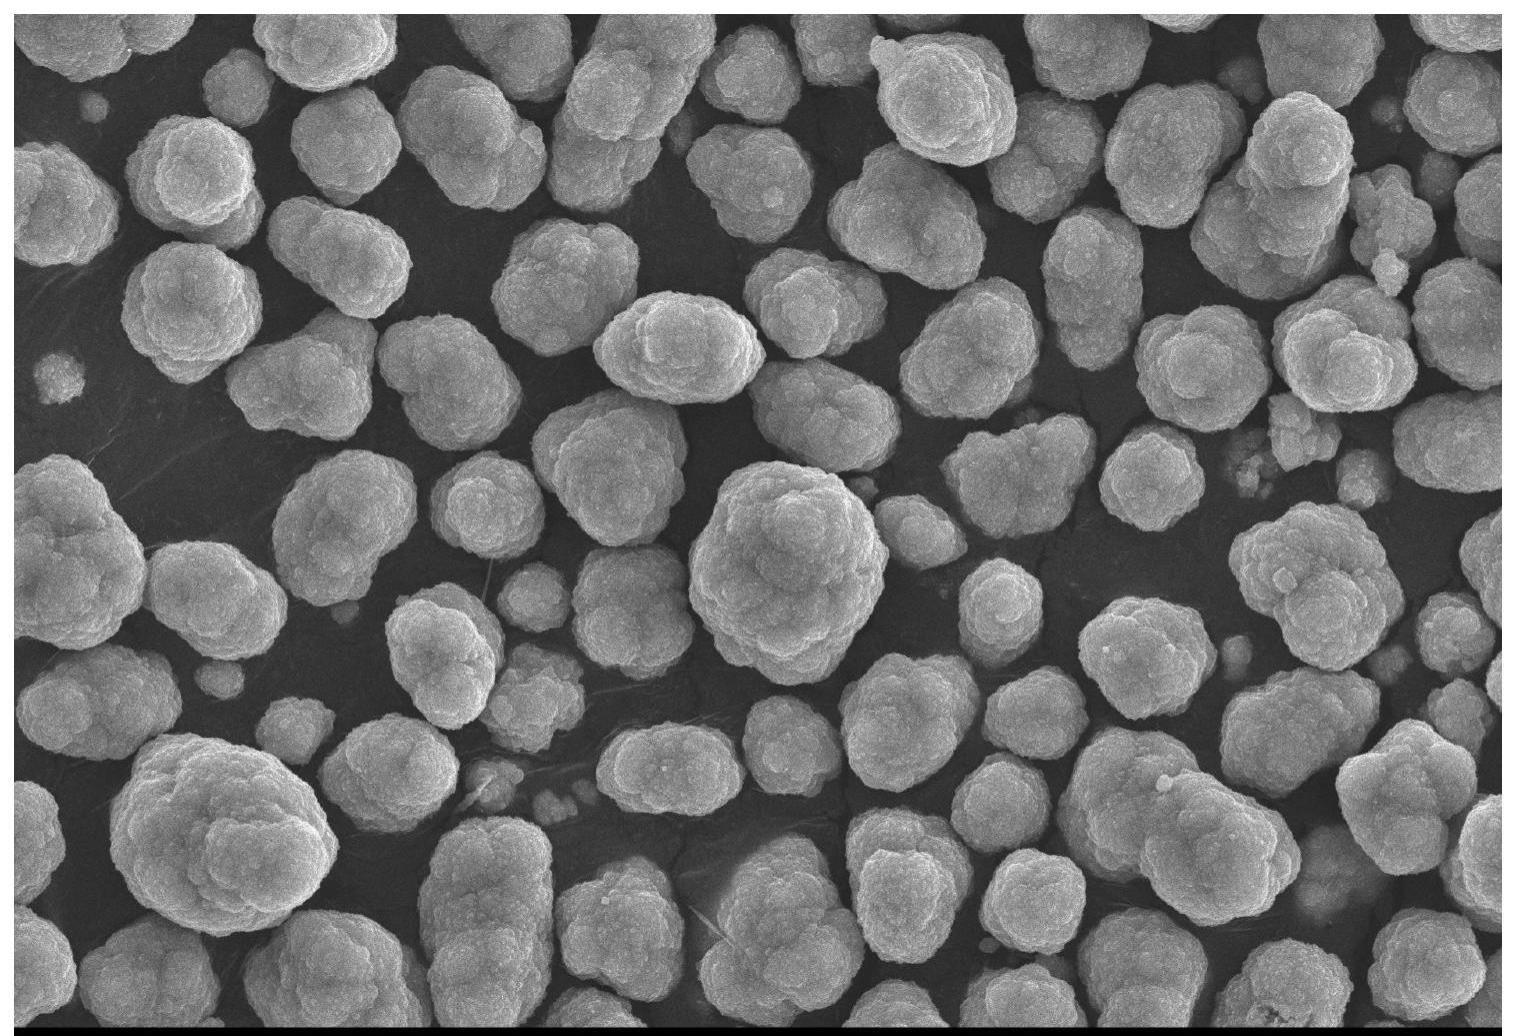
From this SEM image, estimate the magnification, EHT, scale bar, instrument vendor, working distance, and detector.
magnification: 2.5 K X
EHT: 20 kV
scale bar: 10000 nm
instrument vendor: JEOL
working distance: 10 mm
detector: SED